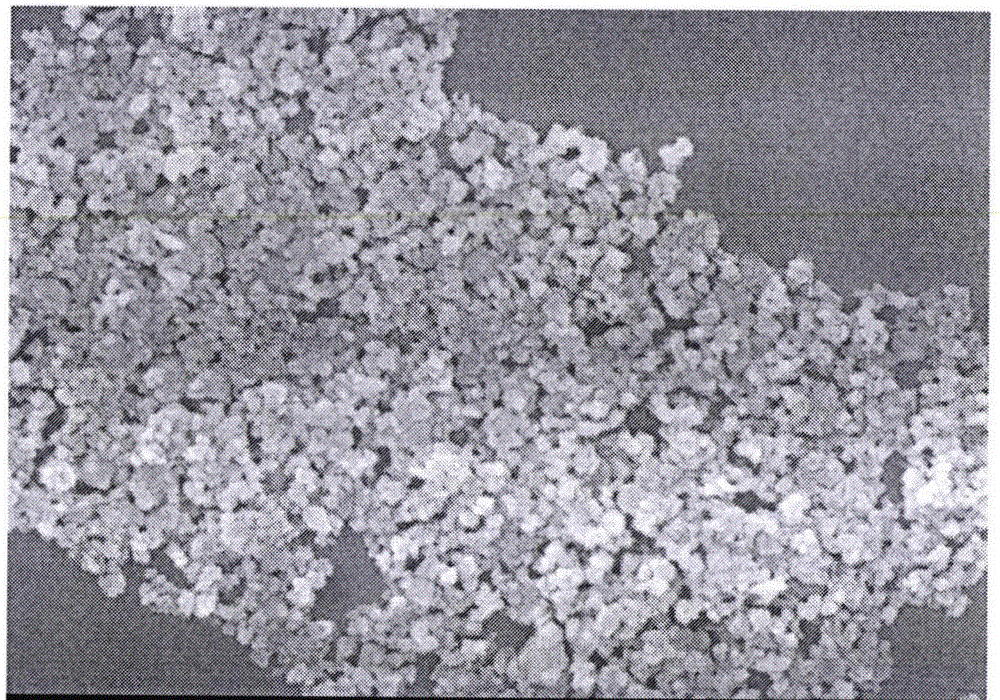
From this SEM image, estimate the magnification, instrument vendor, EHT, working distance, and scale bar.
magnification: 20 K X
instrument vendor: JEOL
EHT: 5 kV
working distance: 8.3 mm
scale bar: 1000 nm